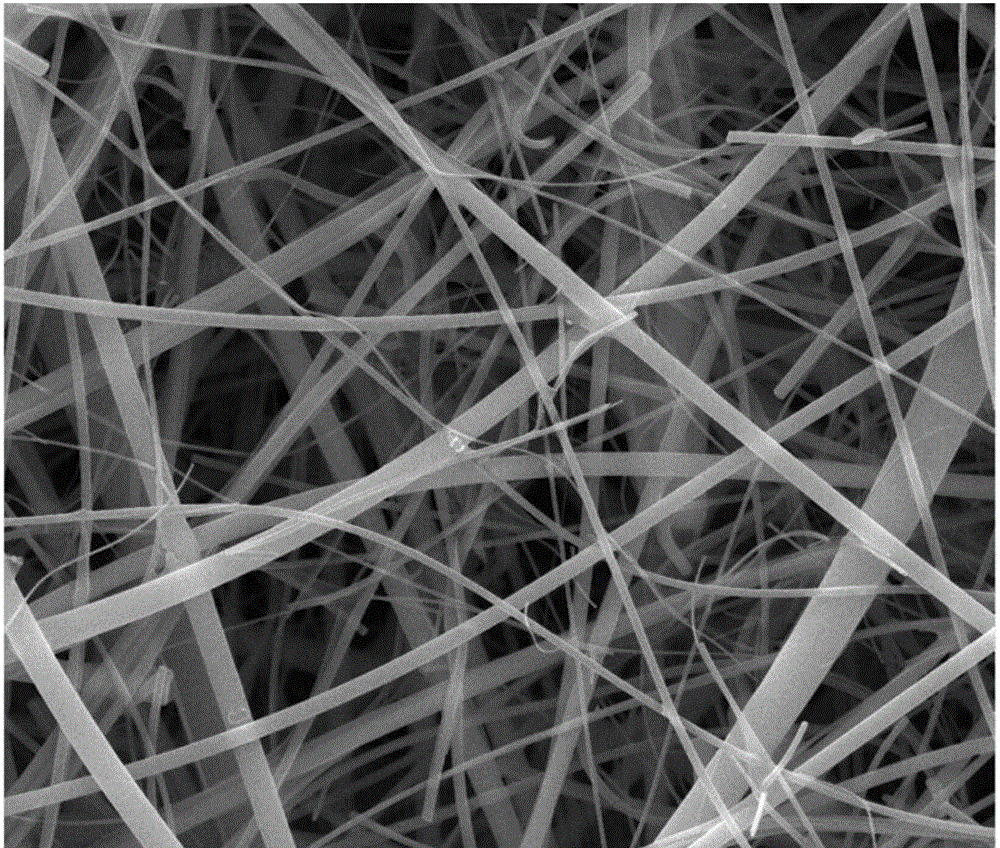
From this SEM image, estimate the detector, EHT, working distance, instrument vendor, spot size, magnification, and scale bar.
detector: ETD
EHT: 25 kV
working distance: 10.1 mm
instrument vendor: FEI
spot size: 2.5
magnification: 5 K X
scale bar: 20000 nm